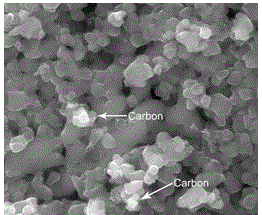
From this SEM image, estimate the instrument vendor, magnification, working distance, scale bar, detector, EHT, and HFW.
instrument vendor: FEI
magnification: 100 K X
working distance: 5.6 mm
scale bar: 500 nm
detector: ETD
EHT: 20 kV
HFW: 2.98 µm